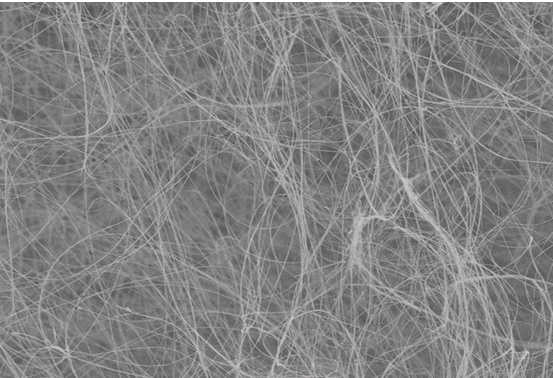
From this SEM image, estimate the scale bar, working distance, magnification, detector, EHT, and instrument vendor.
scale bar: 50000 nm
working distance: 8.3 mm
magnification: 1 K X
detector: SE(M)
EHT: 5 kV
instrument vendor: Hitachi